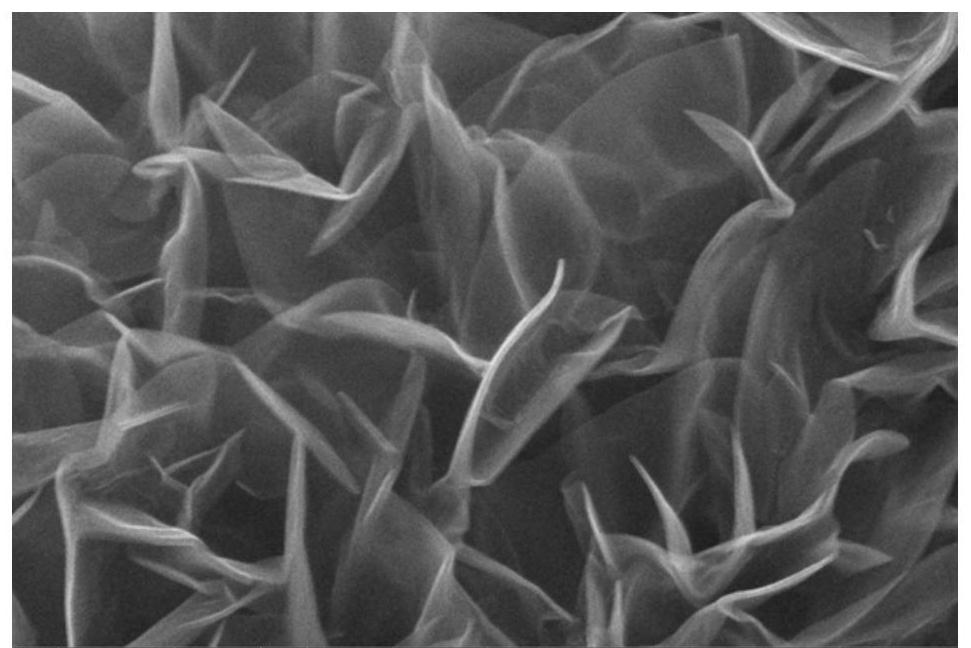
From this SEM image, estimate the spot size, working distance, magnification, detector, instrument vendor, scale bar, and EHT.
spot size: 3.5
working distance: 10.8 mm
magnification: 120 K X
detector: ETD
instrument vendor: FEI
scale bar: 1000 nm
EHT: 25 kV